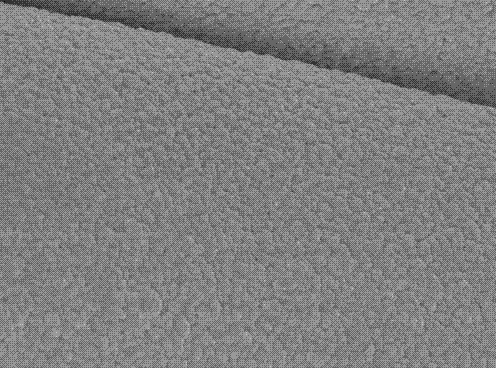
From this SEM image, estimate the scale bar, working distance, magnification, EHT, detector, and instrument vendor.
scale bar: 1000 nm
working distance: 7.7 mm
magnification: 10 K X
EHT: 10 kV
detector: SEI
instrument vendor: JEOL